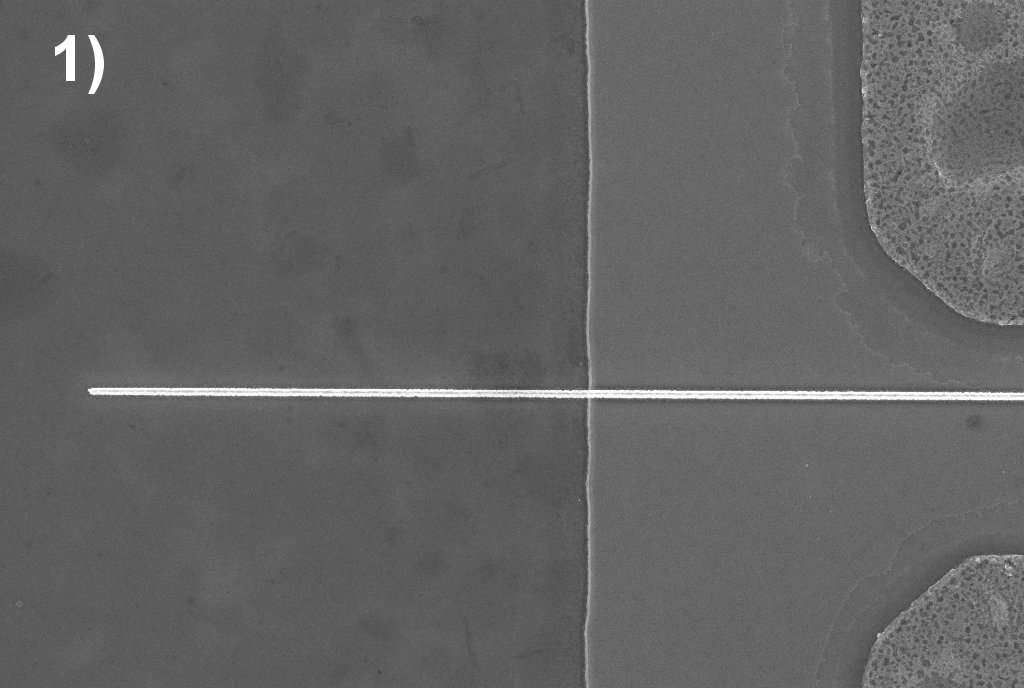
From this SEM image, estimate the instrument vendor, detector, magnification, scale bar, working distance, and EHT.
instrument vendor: Zeiss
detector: InLens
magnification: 6.22 K X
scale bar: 1000 nm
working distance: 4.7 mm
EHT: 5 kV